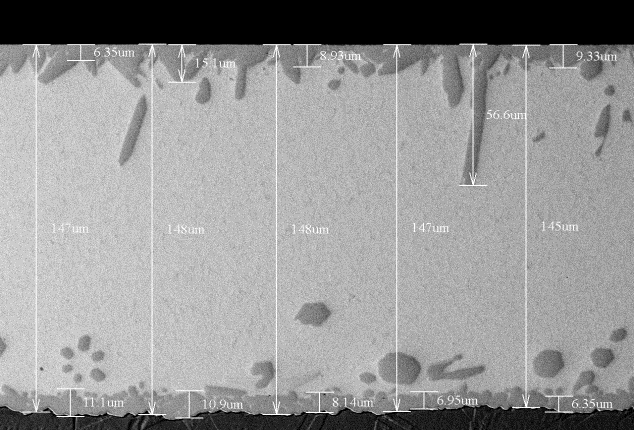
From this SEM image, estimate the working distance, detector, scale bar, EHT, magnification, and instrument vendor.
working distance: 6.9 mm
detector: BSE3D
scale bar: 100000 nm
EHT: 15 kV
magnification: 0.5 K X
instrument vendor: Hitachi